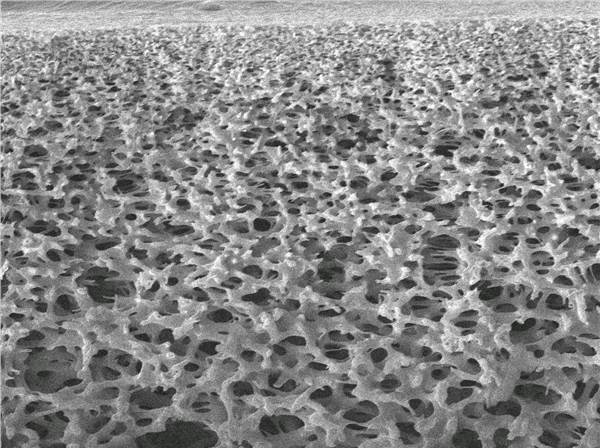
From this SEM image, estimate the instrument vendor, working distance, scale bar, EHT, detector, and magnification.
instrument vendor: JEOL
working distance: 12.4 mm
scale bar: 10000 nm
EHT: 15 kV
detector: SEI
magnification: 1 K X